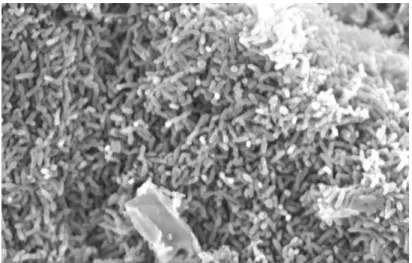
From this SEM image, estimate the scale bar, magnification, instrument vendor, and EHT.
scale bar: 5000 nm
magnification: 4 K X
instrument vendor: JEOL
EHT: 10 kV